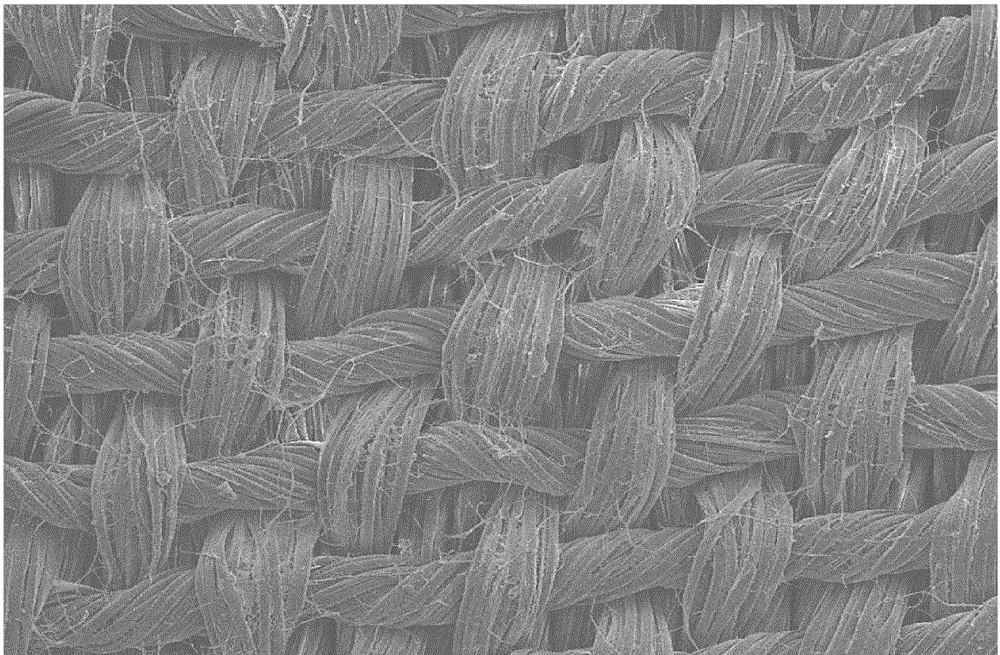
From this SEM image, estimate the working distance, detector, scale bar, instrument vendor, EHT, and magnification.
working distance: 10 mm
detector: SEI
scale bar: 100000 nm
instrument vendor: JEOL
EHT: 15 kV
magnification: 0.1 K X